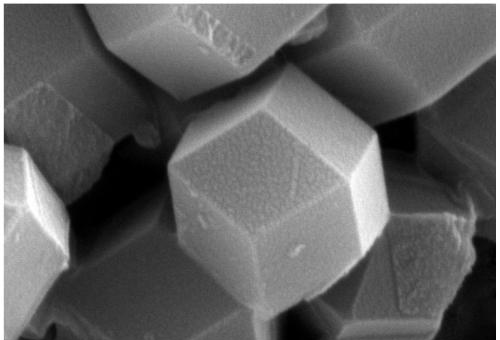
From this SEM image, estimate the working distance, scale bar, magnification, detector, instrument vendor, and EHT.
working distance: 7.7 mm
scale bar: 200 nm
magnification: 50 K X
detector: SE2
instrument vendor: Zeiss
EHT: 10 kV